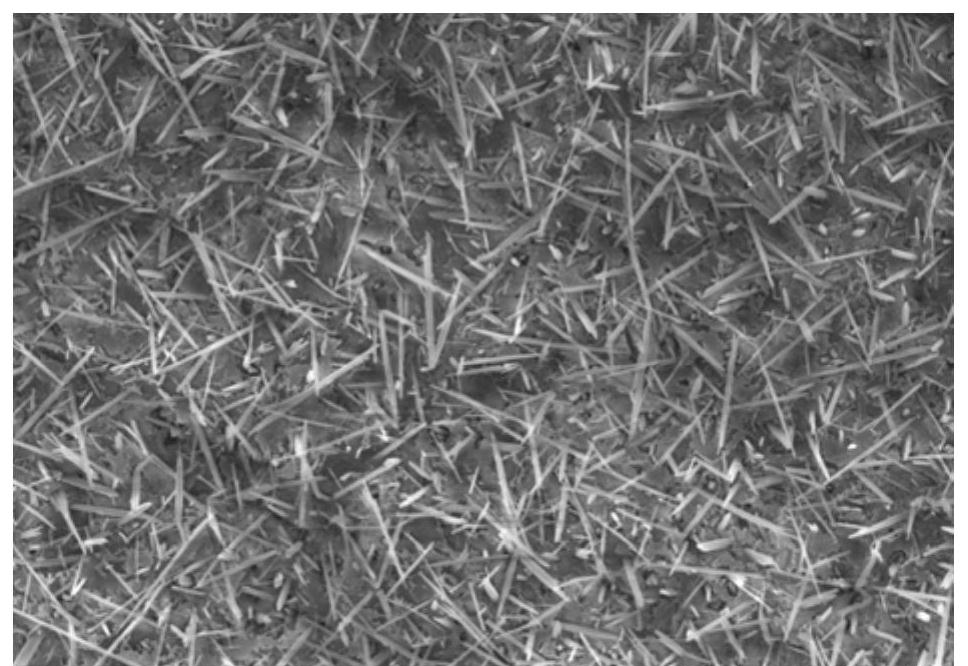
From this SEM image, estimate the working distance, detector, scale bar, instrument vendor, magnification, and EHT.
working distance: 11.6 mm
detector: SE(UL)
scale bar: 20000 nm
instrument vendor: Hitachi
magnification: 5 K X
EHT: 10 kV